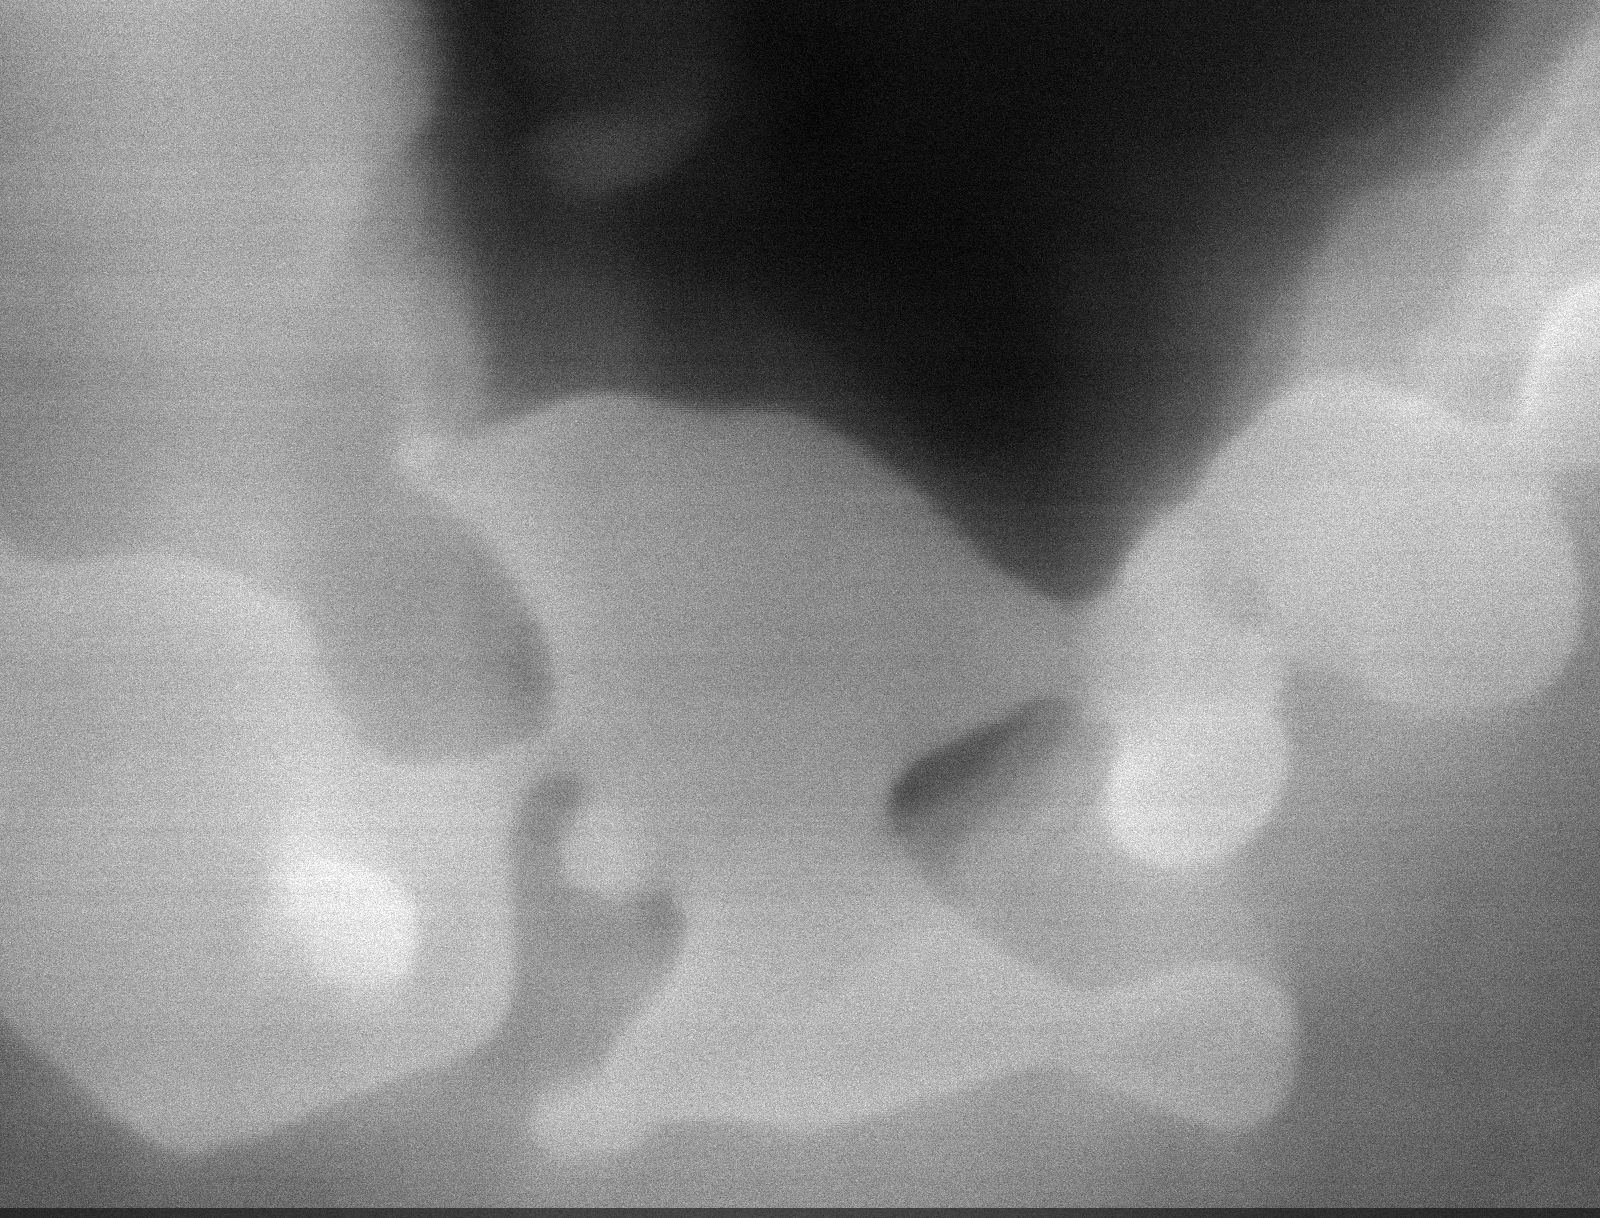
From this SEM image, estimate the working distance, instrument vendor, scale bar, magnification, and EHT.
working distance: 6 mm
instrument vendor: Tescan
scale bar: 100 nm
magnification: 200 K X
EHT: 12 kV